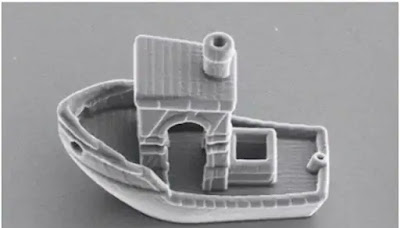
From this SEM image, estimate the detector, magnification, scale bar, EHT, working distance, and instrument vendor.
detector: ETD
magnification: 3.5 K X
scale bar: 20000 nm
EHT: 5 kV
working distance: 12.9 mm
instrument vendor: Thermo